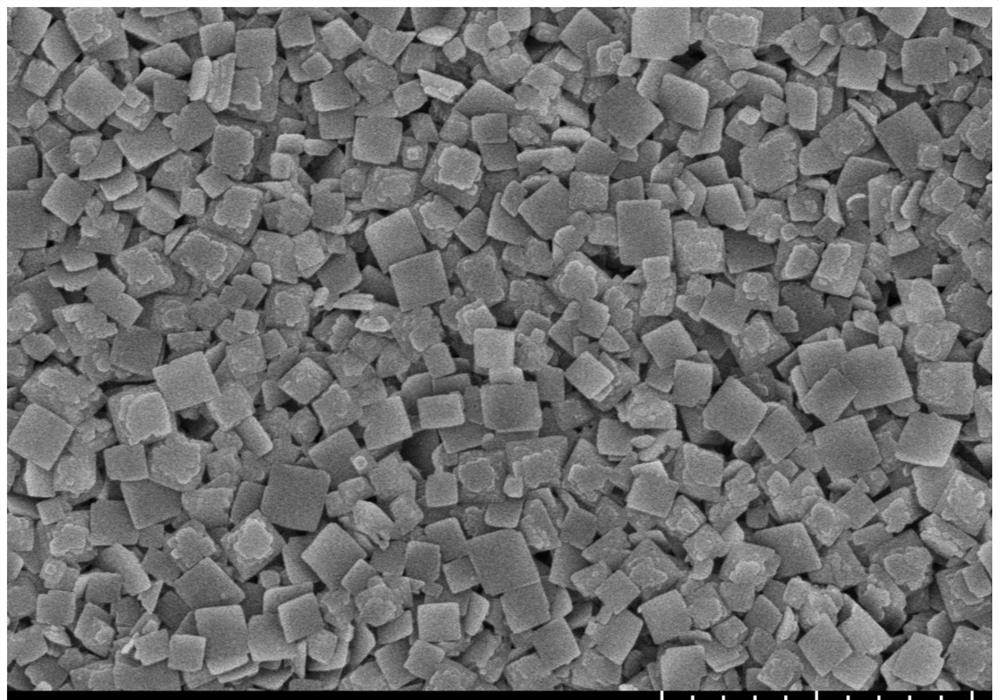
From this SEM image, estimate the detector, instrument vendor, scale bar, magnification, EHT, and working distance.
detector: SE(UL)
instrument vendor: Hitachi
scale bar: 2000 nm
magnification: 20 K X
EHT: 5 kV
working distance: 9.7 mm